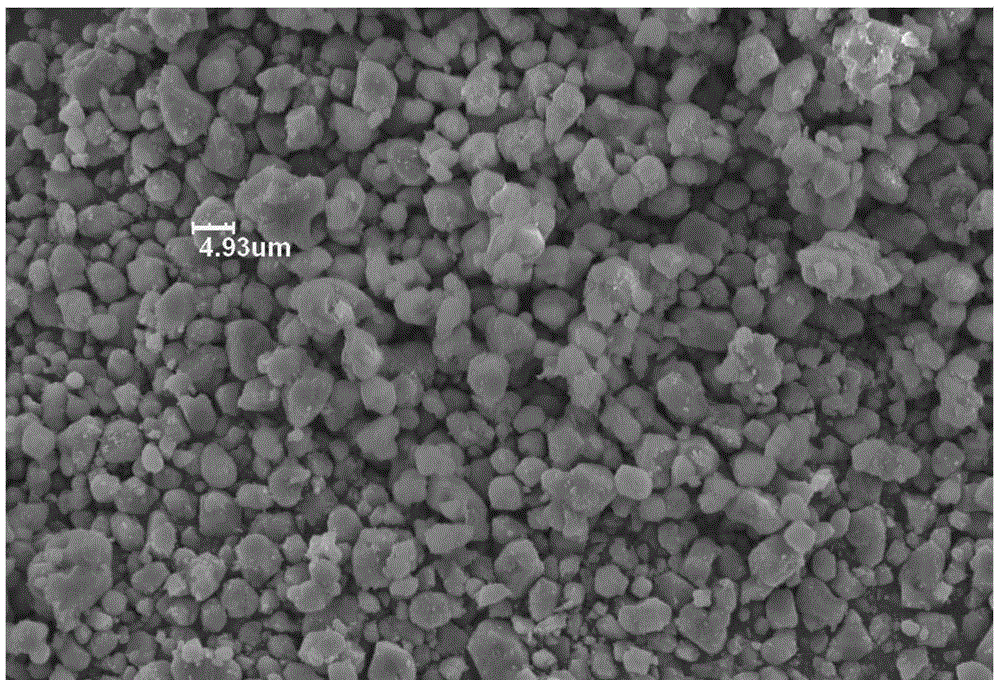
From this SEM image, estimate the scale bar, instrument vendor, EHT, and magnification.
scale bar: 10000 nm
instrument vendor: other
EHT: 19 kV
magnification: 1 K X